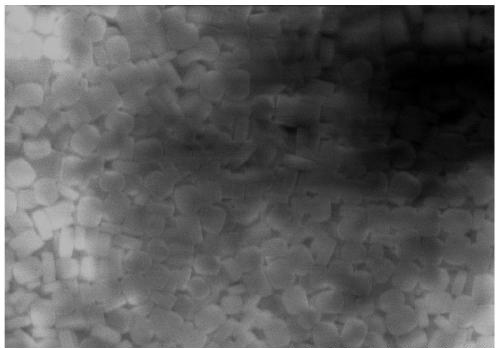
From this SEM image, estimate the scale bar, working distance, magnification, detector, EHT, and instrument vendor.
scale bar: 5000 nm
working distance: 7.2 mm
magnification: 10 K X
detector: SE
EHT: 15 kV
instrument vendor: Hitachi